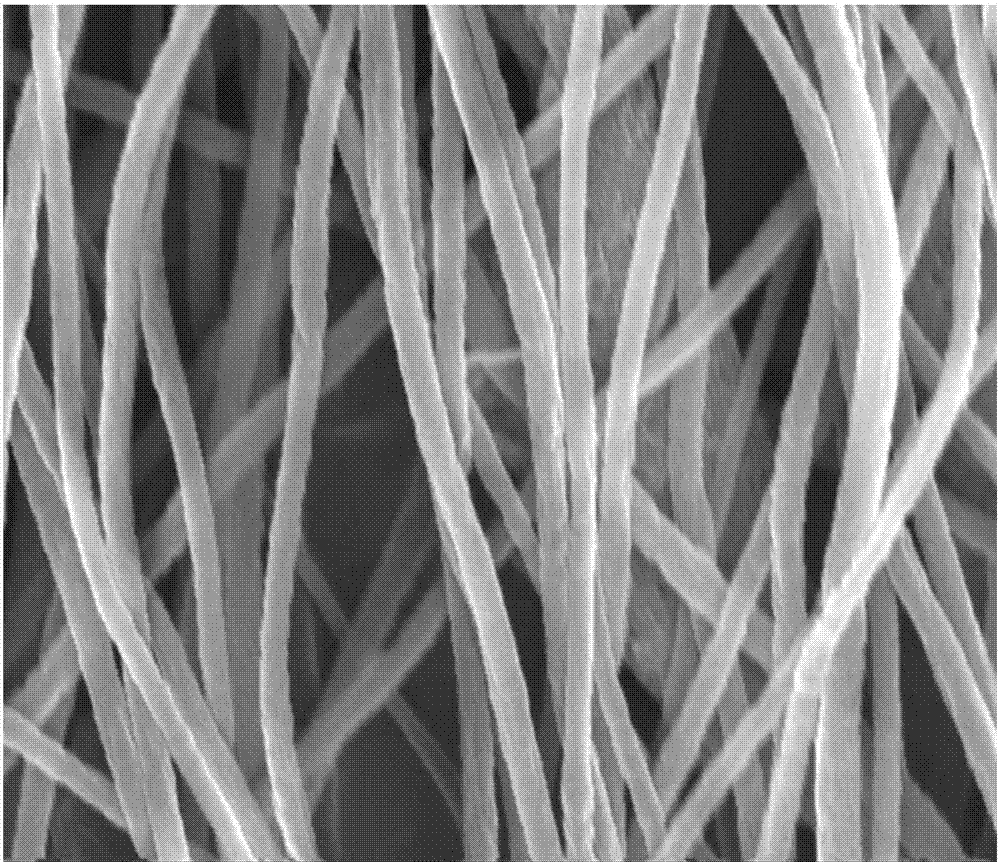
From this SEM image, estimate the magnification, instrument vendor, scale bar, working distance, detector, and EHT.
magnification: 80 K X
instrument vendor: FEI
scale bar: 1000 nm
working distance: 5.1 mm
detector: TLD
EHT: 10 kV